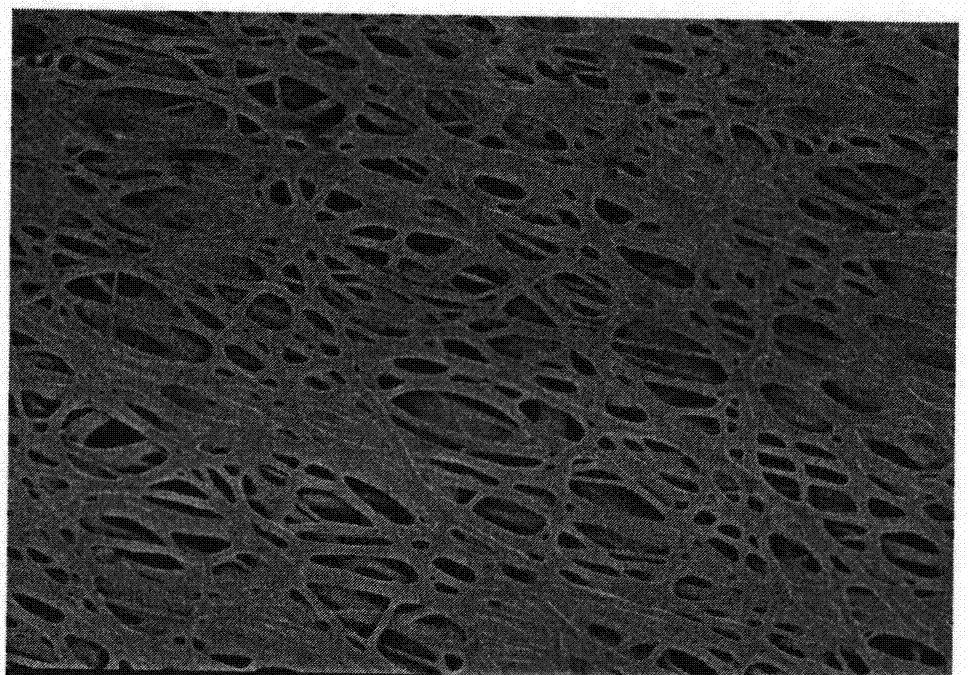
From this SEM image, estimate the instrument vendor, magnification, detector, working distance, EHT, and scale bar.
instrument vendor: Hitachi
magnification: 2 K X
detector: SE(U)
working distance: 8.7 mm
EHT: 1 kV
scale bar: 20000 nm